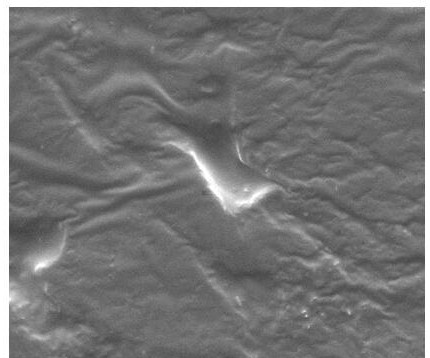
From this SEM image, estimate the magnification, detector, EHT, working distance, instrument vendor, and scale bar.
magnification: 5 K X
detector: ETD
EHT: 10 kV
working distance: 9.1 mm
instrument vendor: FEI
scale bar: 30000 nm